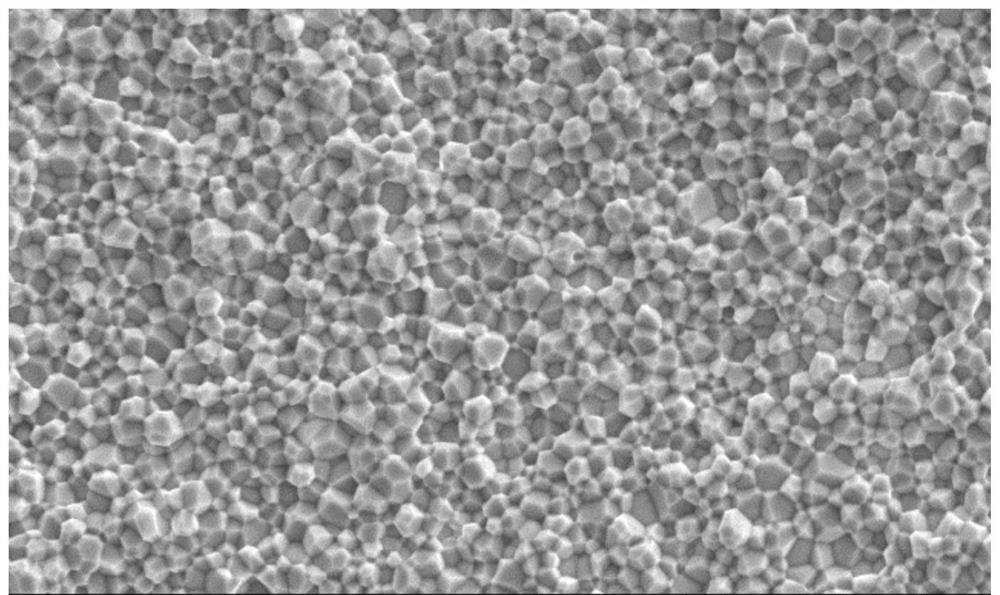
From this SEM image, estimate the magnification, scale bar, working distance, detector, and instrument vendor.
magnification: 1 K X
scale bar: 50000 nm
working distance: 12.3 mm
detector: SE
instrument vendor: Hitachi